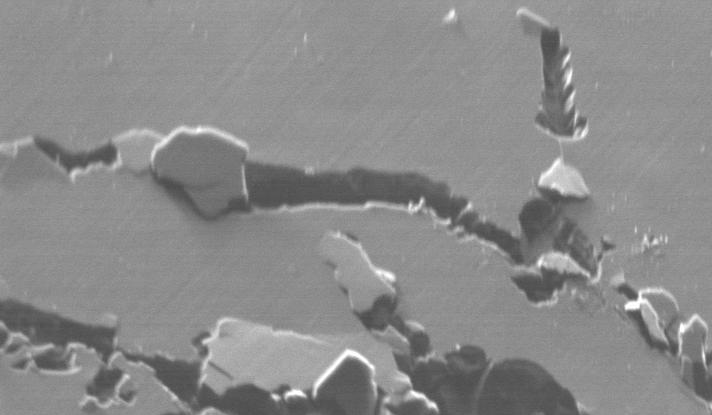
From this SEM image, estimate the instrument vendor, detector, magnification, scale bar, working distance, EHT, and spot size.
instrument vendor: FEI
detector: BSE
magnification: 2 K X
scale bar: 20000 nm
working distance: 17 mm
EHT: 20 kV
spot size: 5.5